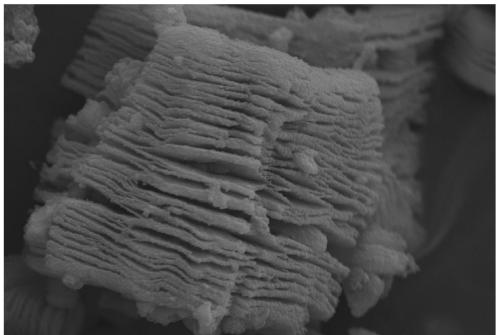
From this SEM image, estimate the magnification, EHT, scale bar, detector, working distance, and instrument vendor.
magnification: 5 K X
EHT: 15 kV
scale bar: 2000 nm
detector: SE2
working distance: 8.4 mm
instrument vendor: Zeiss